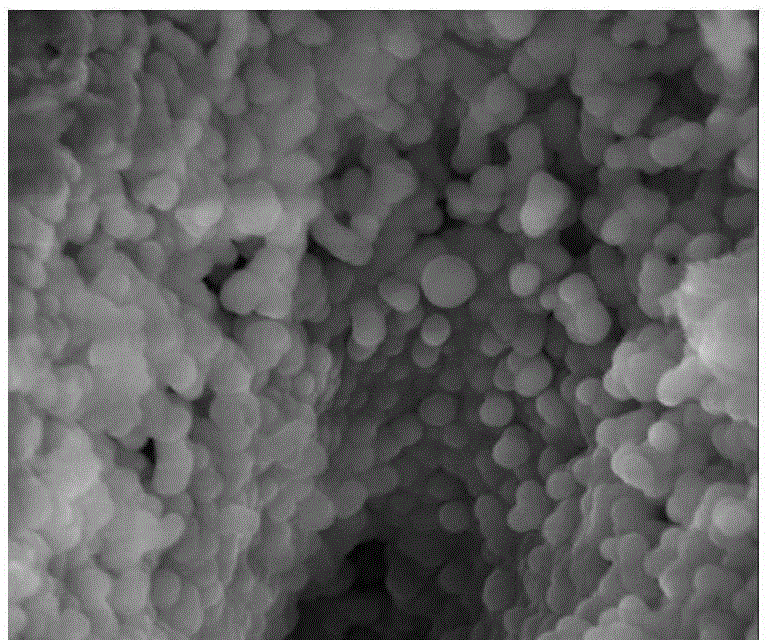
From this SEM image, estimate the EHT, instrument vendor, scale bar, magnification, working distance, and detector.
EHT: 10 kV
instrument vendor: FEI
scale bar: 1000 nm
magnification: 50 K X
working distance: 5.3 mm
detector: TLD